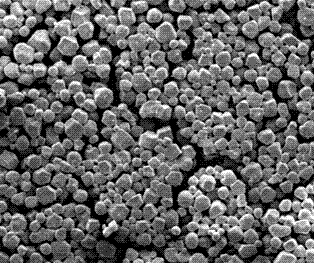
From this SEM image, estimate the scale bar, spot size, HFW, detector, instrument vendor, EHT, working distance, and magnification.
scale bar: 50000 nm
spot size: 2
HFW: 298 µm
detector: ETD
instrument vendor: FEI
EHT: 10 kV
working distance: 6.8 mm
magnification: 0.5 K X